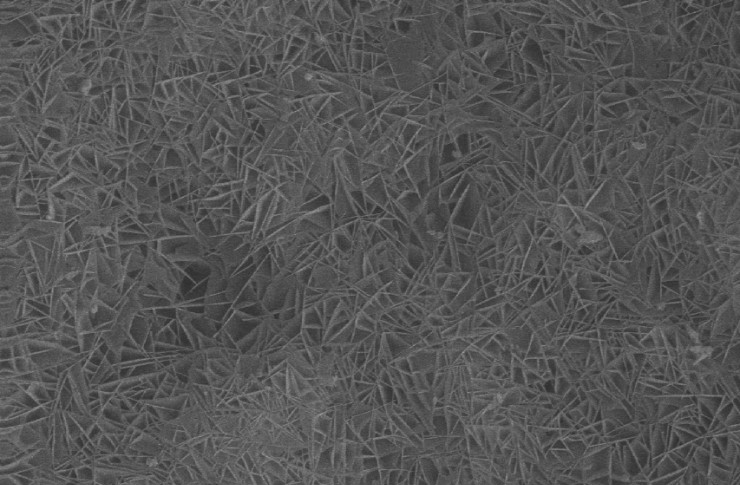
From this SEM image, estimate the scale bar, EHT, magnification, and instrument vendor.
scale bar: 3330 nm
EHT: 20 kV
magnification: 3 K X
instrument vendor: JEOL